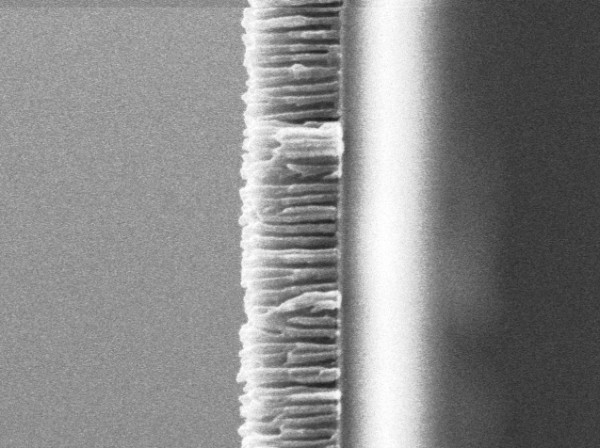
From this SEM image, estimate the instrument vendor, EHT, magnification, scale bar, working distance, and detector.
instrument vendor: JEOL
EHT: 5 kV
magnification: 37 K X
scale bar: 100 nm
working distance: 6 mm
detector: SEI